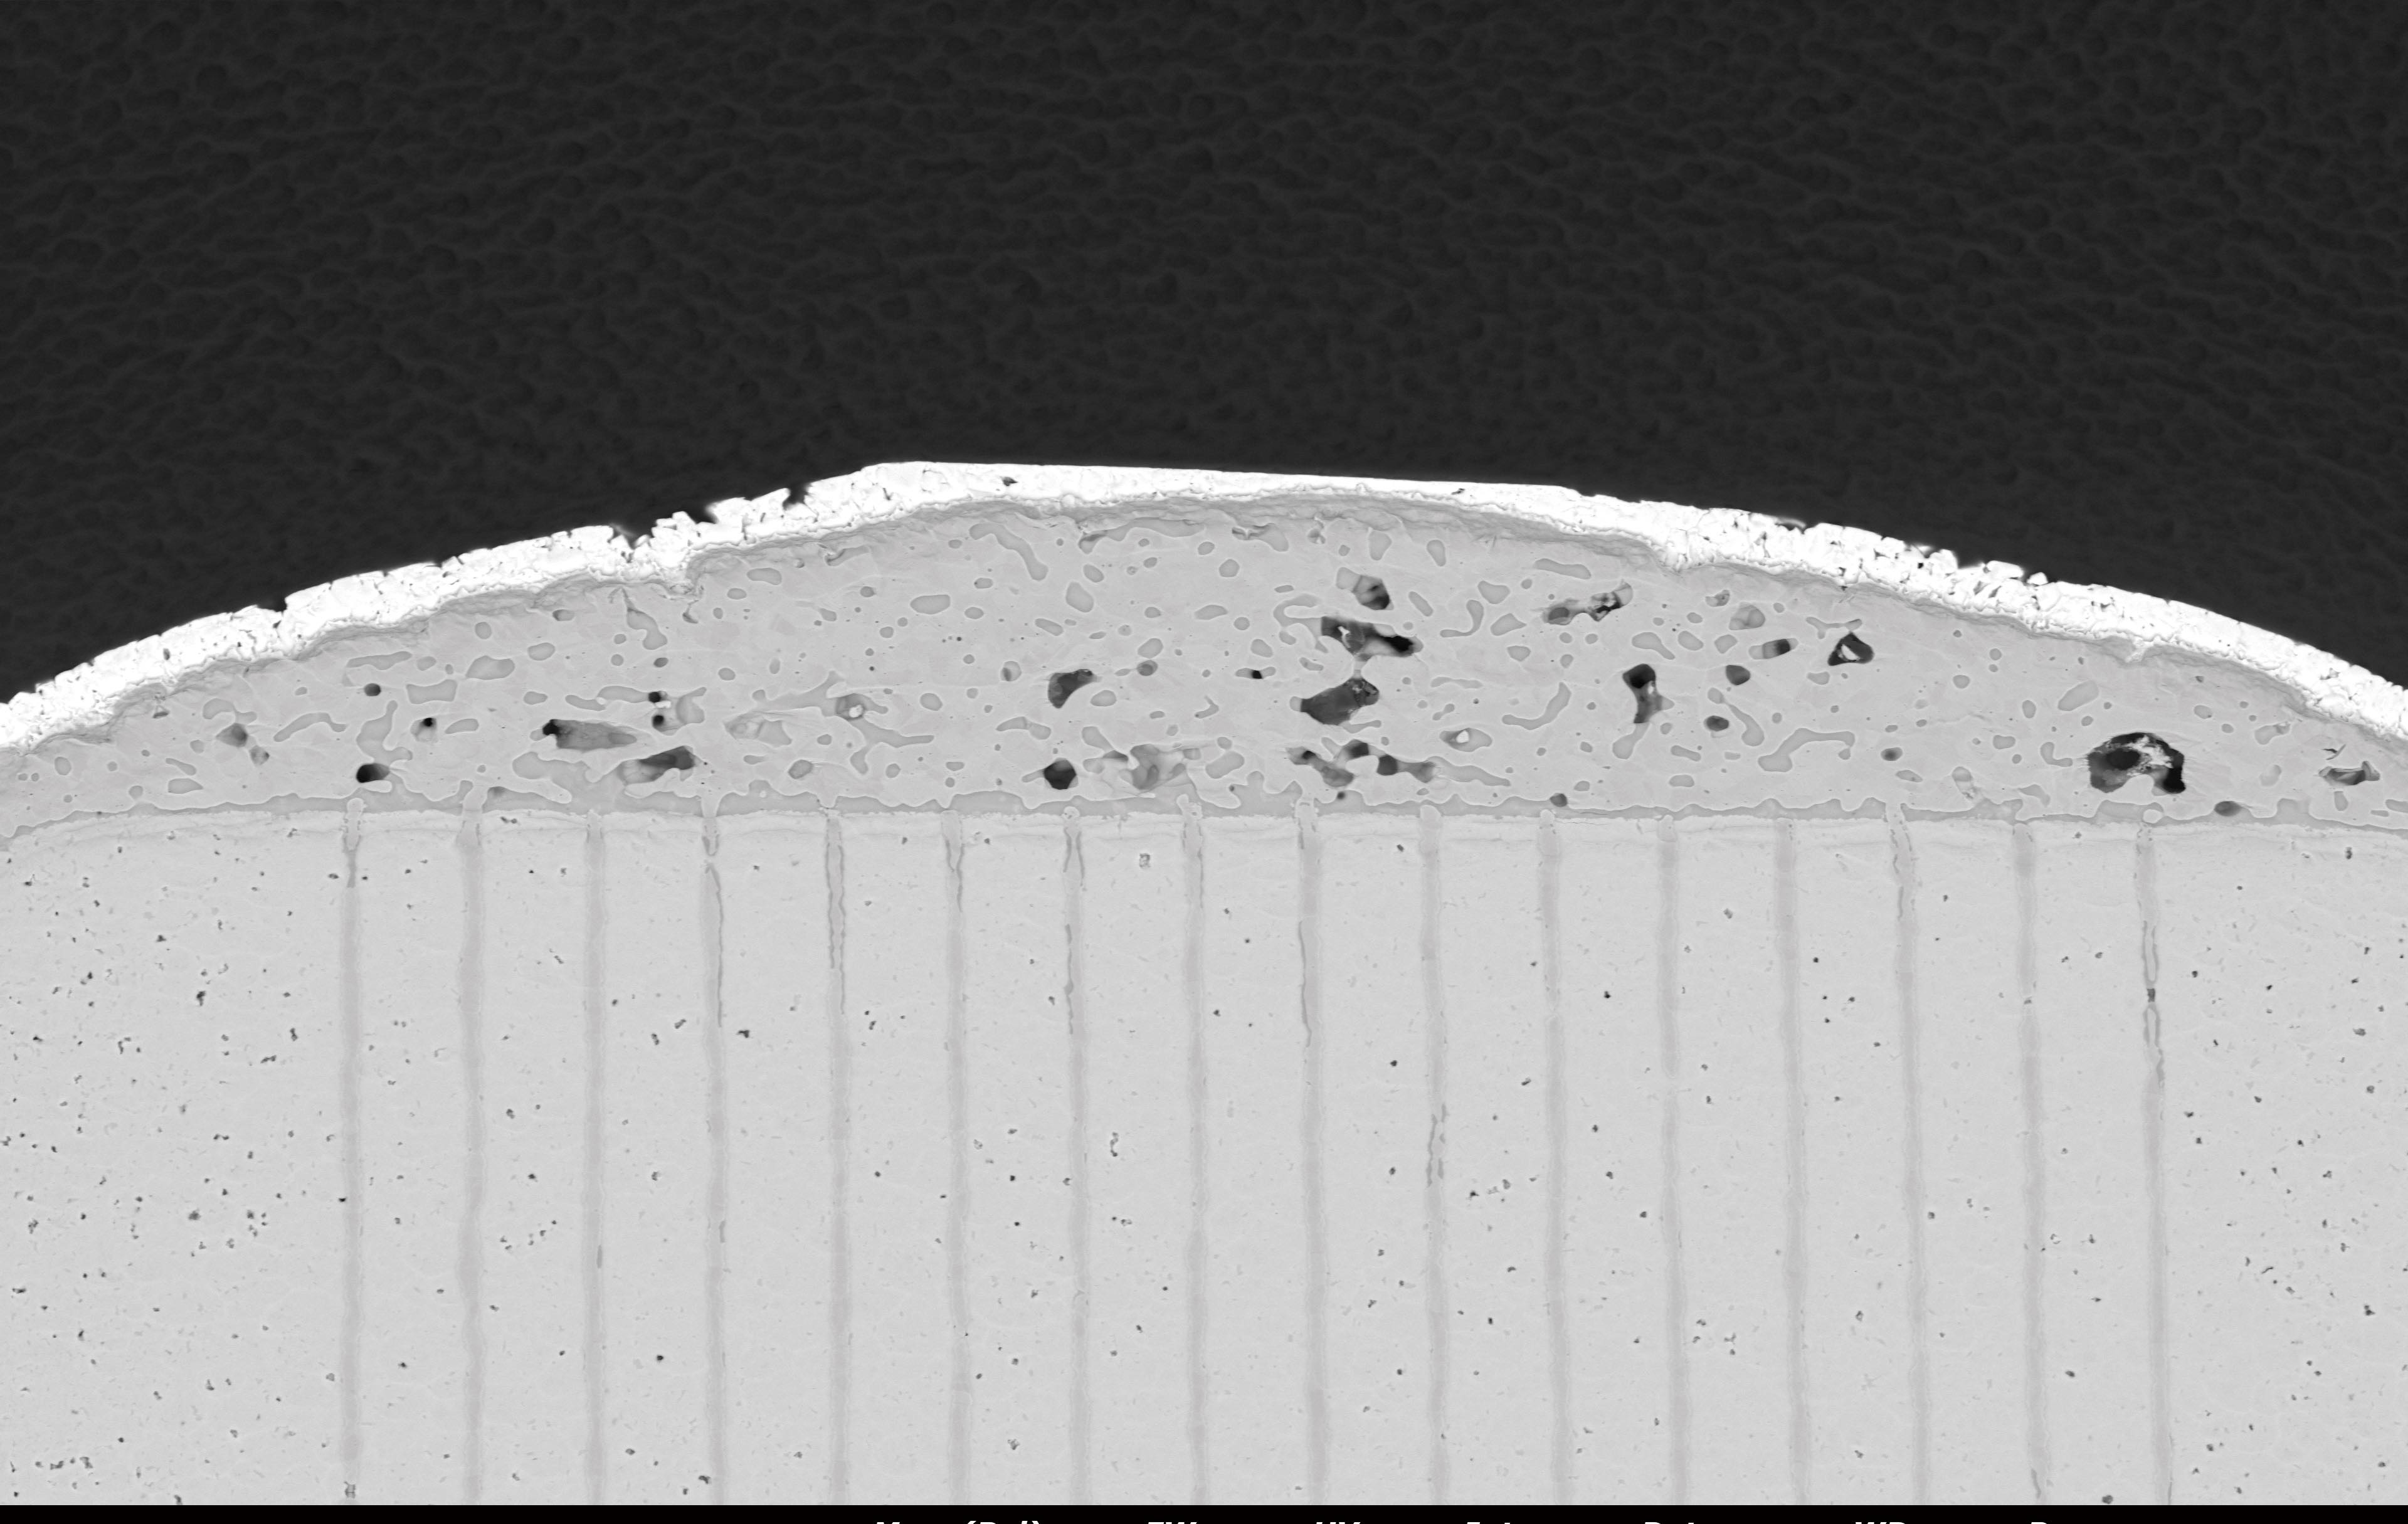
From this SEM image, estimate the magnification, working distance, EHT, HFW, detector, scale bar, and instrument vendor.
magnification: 0.5 K X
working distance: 9.168 mm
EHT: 15 kV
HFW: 254 µm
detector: BSD Full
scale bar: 80000 nm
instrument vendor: other